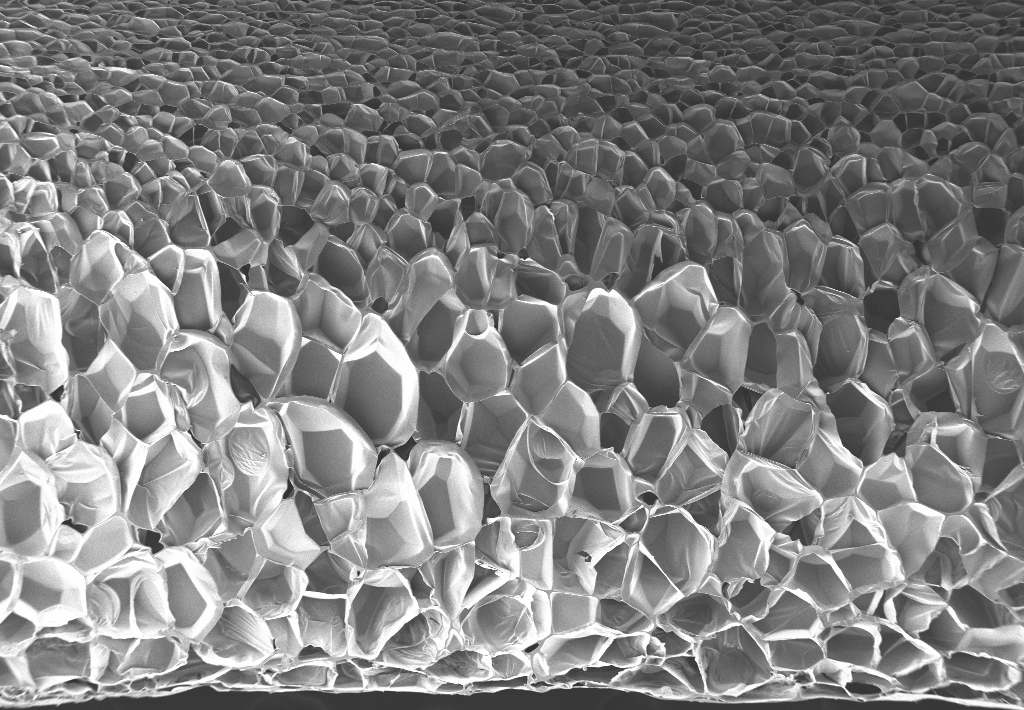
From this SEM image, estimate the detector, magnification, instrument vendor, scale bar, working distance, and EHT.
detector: SE2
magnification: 0.05 K X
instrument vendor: Zeiss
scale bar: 200000 nm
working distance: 13.4 mm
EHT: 3 kV